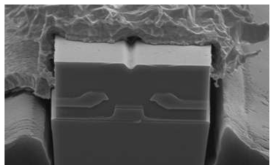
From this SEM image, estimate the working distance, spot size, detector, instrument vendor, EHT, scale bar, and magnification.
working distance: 13.4 mm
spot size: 4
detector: SE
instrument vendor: FEI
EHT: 10 kV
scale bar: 2000 nm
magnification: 7.597 K X